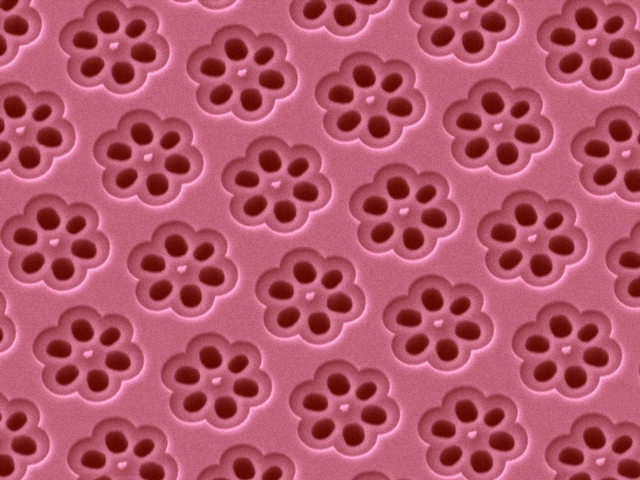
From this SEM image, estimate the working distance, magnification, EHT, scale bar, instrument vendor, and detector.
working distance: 12.6 mm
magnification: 1.8 K X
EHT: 10 kV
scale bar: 10000 nm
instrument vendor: JEOL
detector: LEI